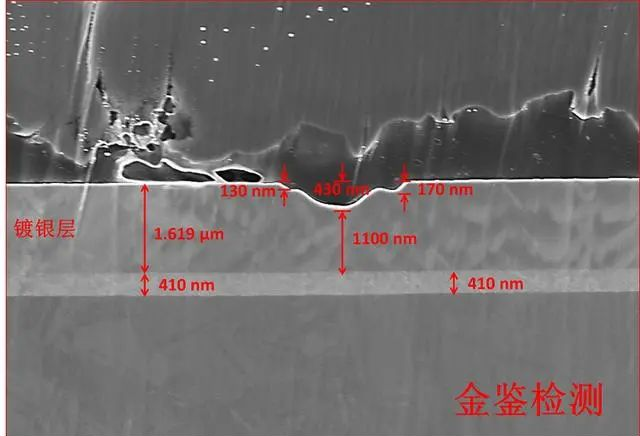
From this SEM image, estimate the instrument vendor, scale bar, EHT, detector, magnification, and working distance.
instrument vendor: Zeiss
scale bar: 1000 nm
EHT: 5 kV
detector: InLens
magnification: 10 K X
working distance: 4.3 mm